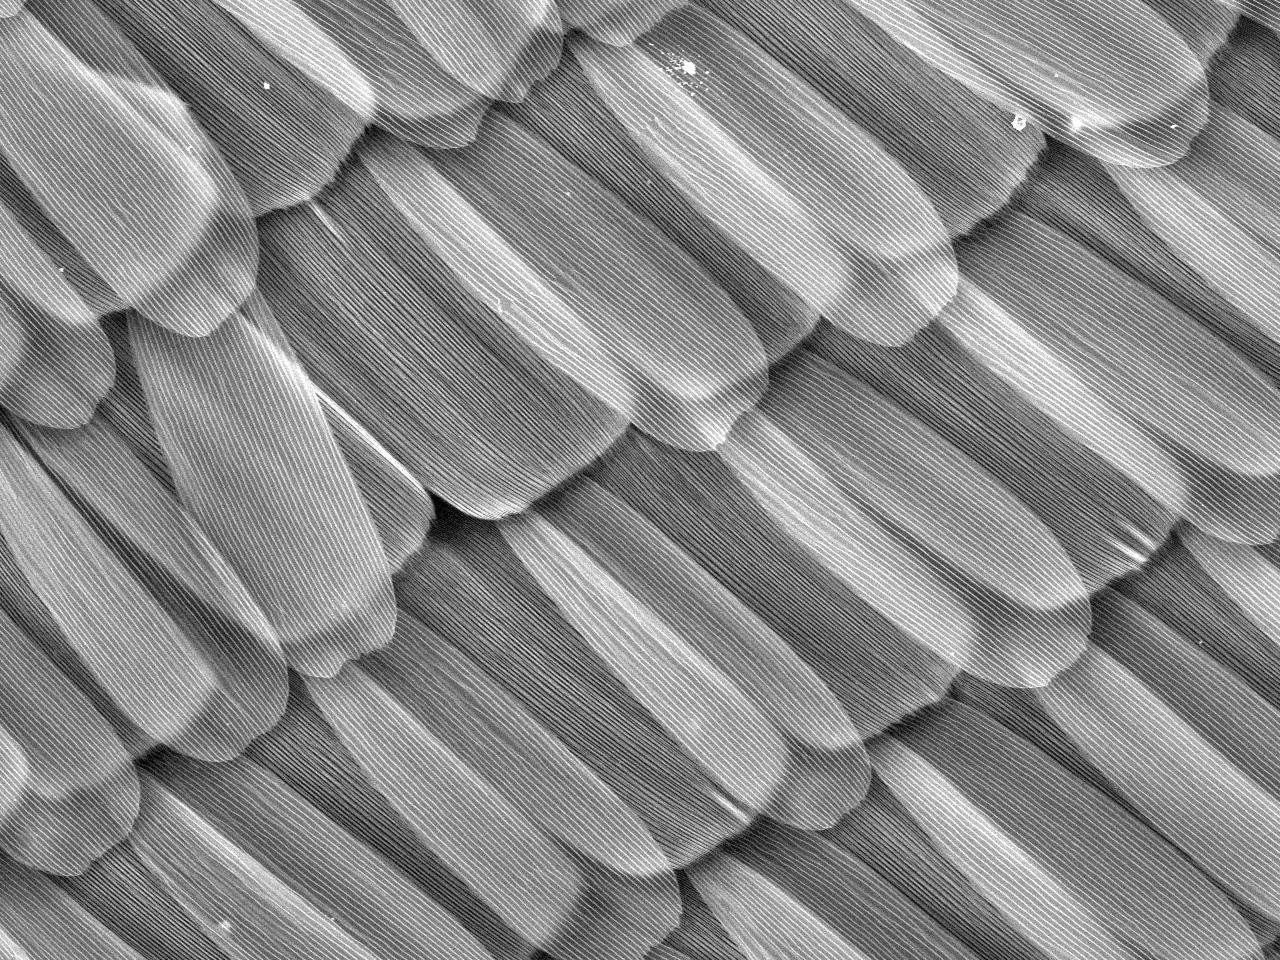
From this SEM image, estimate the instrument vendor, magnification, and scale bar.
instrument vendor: Hitachi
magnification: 0.4 K X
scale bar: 200000 nm